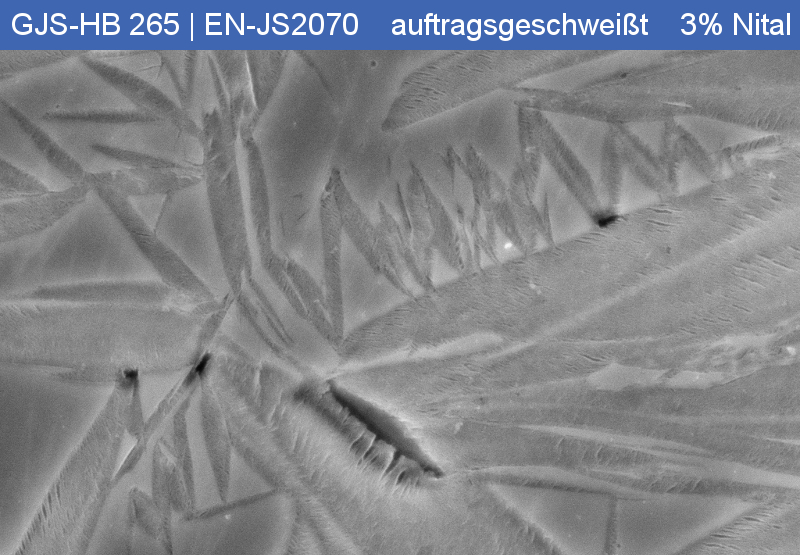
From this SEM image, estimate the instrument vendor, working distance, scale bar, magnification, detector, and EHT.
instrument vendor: Zeiss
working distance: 5 mm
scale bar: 1000 nm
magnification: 10 K X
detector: InLens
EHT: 15 kV